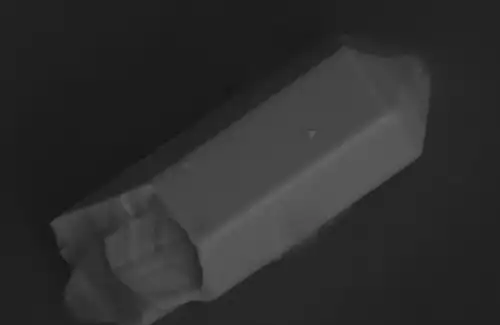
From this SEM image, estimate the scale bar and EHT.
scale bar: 10000 nm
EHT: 25 kV